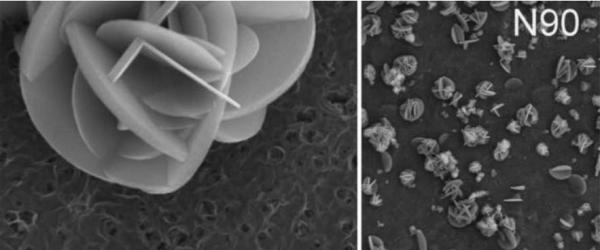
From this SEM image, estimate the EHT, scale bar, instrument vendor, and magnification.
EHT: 15 kV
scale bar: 50000 nm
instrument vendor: JEOL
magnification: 0.5 K X